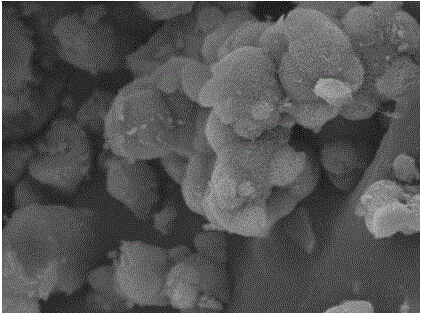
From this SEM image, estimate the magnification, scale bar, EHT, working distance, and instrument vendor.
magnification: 3.02 K X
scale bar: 10000 nm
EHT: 15 kV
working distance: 14.69 mm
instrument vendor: Tescan